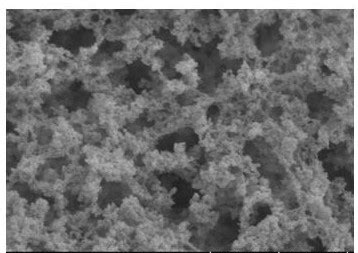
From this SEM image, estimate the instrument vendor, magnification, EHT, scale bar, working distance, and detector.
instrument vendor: Hitachi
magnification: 50 K X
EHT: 3 kV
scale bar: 1000 nm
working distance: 8.2 mm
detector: SE(UL)